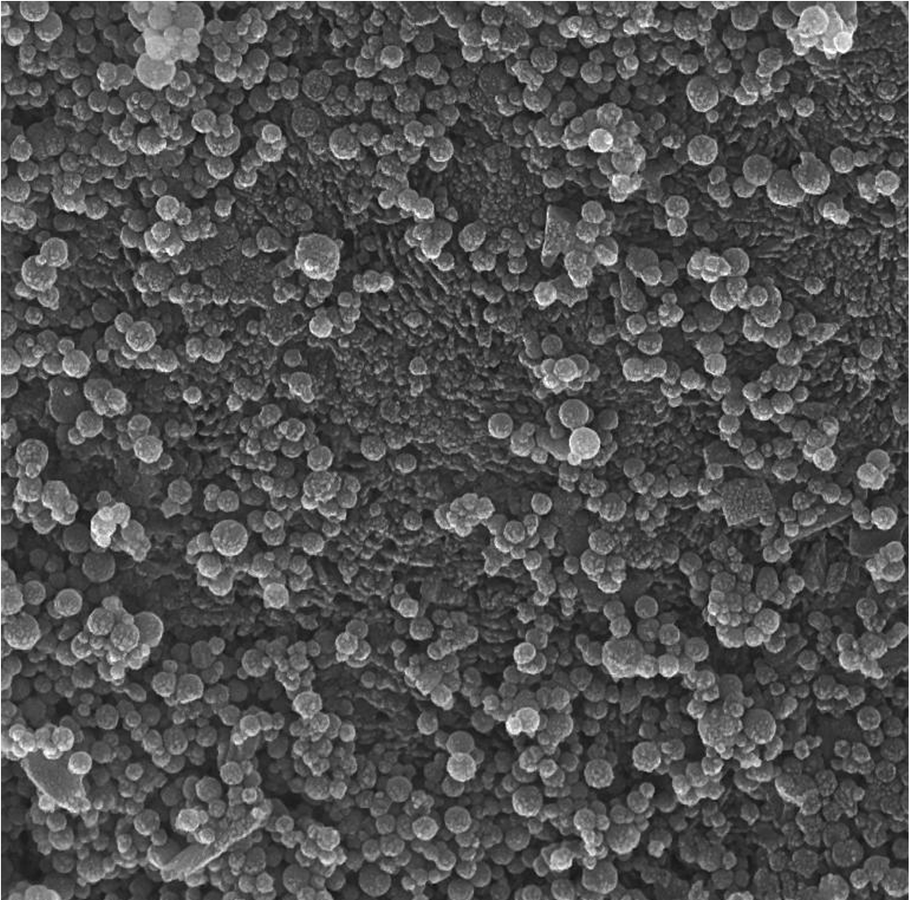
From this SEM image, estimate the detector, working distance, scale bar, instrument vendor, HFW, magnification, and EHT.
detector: In-Beam SE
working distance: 5.39 mm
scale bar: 500 nm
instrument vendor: Tescan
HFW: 2.77 µm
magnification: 75 K X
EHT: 15 kV